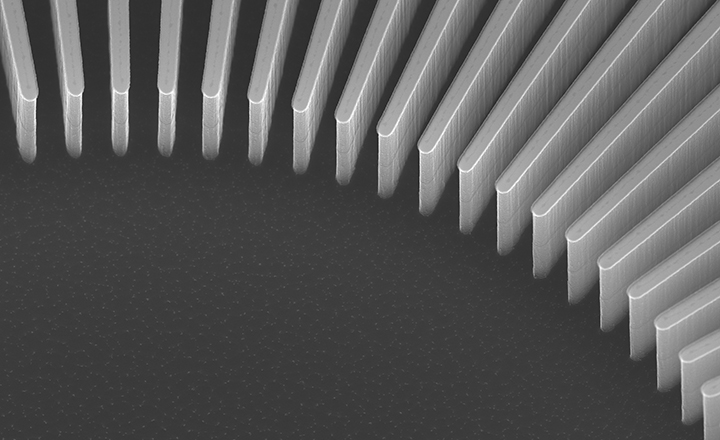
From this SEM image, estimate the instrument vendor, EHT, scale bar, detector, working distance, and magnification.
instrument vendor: Hitachi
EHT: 20 kV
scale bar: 10000 nm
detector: UD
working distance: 8.6 mm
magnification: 4.5 K X